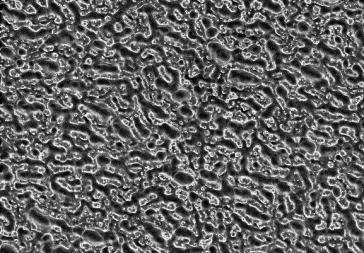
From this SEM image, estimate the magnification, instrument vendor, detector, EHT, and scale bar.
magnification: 5 K X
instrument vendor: Hitachi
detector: SE(UL)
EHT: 2 kV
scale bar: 10000 nm